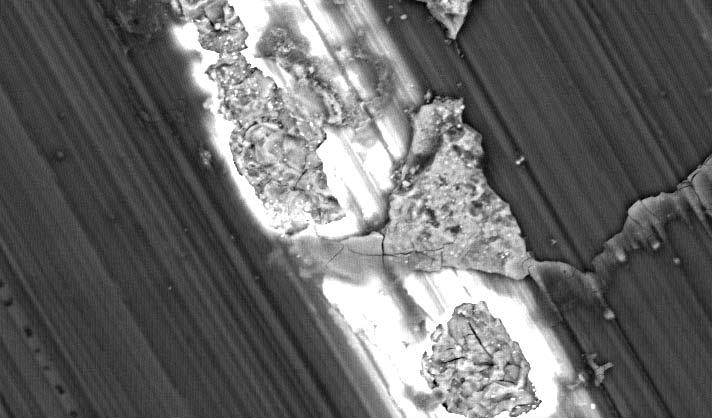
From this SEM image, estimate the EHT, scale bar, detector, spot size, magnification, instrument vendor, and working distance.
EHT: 20 kV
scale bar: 50000 nm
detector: BSE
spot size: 4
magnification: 0.5 K X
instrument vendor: FEI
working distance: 10.6 mm